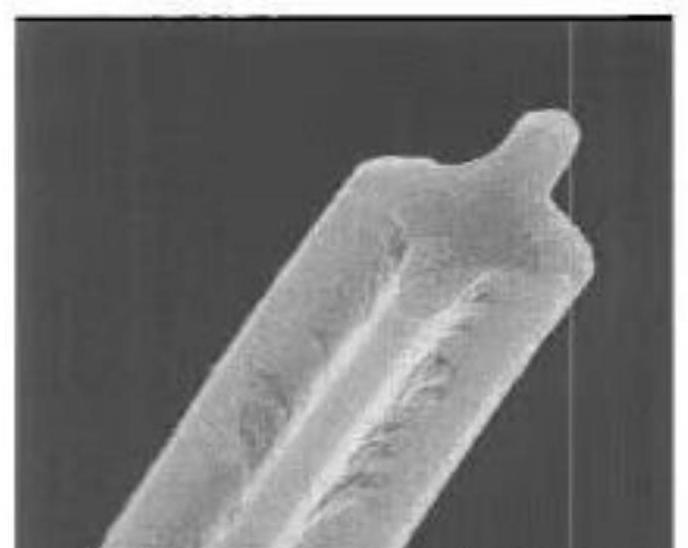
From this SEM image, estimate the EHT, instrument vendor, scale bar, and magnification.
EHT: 18 kV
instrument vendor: JEOL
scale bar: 20000 nm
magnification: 2.5 K X